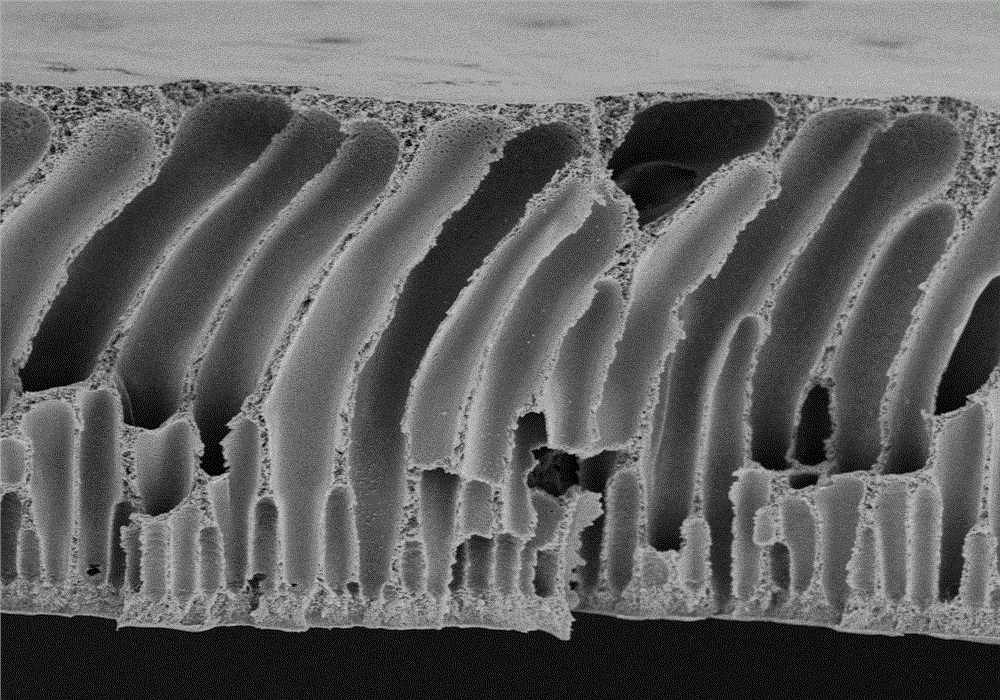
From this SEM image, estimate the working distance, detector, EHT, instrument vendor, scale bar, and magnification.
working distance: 9 mm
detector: SE(M)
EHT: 5 kV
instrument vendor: Hitachi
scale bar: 50000 nm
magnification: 0.6 K X